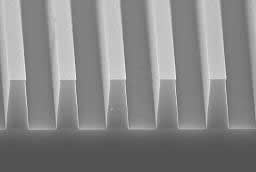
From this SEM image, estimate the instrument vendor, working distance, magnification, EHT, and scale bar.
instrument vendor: JEOL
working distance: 9.5 mm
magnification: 4 K X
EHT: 20 kV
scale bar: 2500 nm